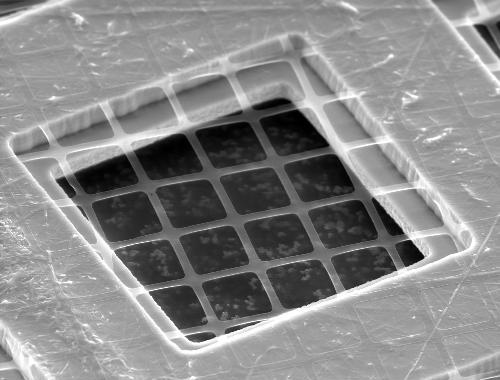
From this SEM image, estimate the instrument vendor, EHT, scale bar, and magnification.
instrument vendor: other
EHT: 25 kV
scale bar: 20000 nm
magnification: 1.9 K X